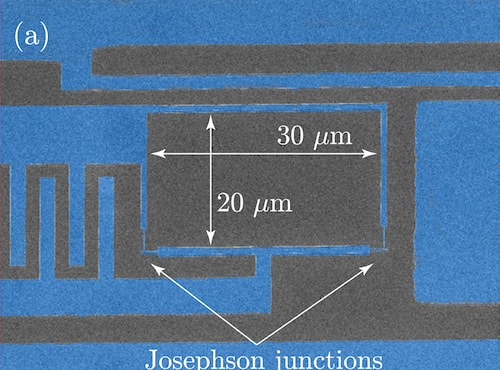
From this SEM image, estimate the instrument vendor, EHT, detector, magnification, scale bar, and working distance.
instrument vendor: JEOL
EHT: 20 kV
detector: LED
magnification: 1.7 K X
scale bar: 10000 nm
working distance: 8.9 mm